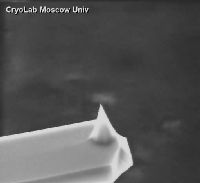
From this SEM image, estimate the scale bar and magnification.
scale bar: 8000 nm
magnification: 0.921 K X